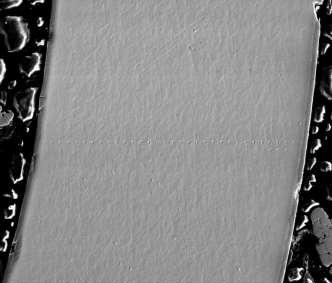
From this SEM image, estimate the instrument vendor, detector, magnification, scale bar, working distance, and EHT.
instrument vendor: FEI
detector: ETD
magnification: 0.18 K X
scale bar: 300000 nm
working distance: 10.1 mm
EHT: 20 kV